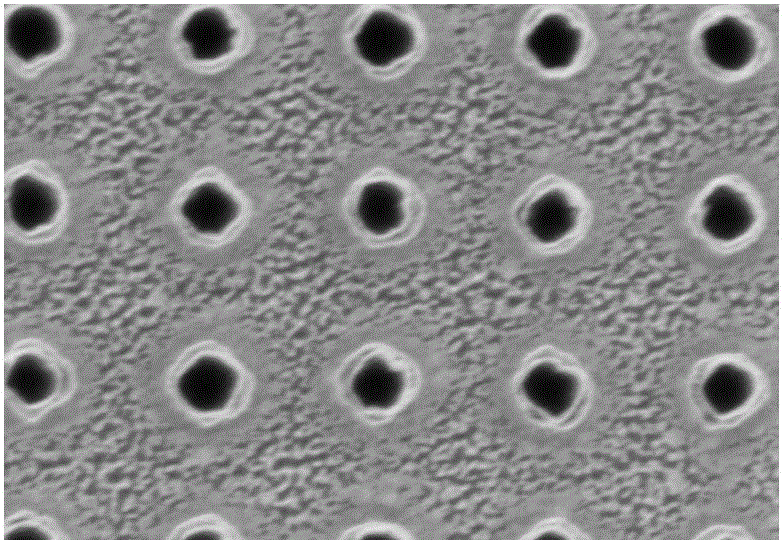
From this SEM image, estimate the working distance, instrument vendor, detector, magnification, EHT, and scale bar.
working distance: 8.1 mm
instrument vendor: Hitachi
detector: SE(UL)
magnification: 70 K X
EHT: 1 kV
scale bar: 500 nm